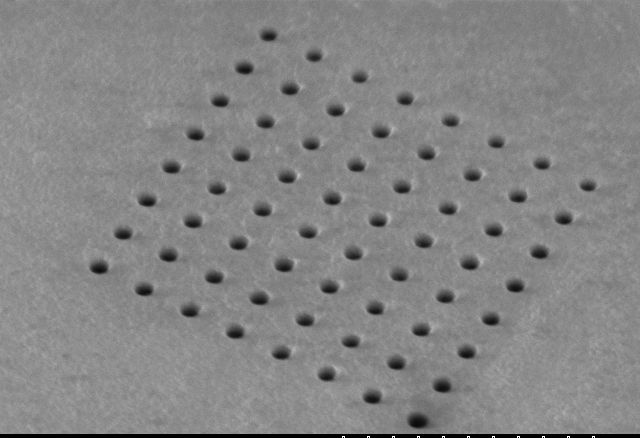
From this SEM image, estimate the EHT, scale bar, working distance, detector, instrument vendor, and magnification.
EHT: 5 kV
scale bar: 10000 nm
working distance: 10.2 mm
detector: SE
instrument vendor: Hitachi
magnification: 5 K X